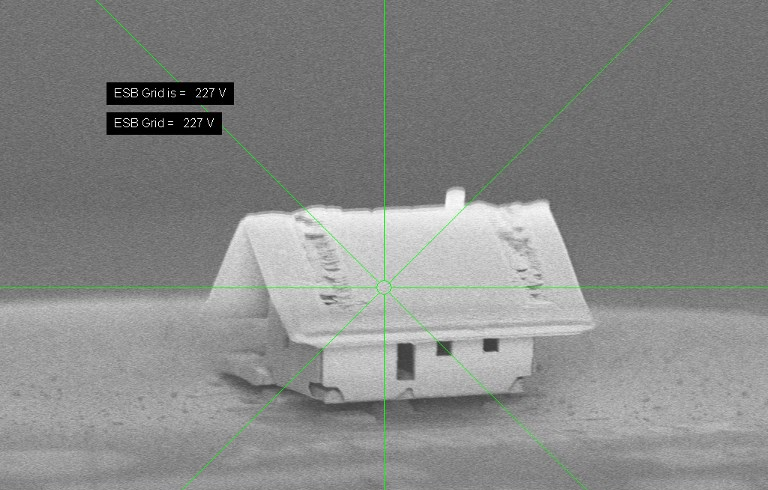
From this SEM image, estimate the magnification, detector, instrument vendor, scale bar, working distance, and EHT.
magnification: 1.76 K X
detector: ESB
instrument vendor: Zeiss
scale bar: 10000 nm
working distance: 4.2 mm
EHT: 0.6 kV